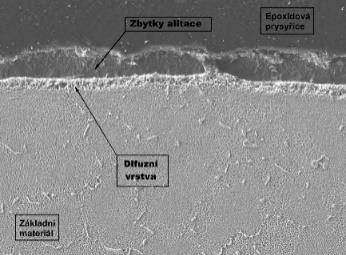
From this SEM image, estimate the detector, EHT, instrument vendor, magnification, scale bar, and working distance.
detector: LEI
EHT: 15 kV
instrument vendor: JEOL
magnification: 0.5 K X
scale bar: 10000 nm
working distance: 7.9 mm